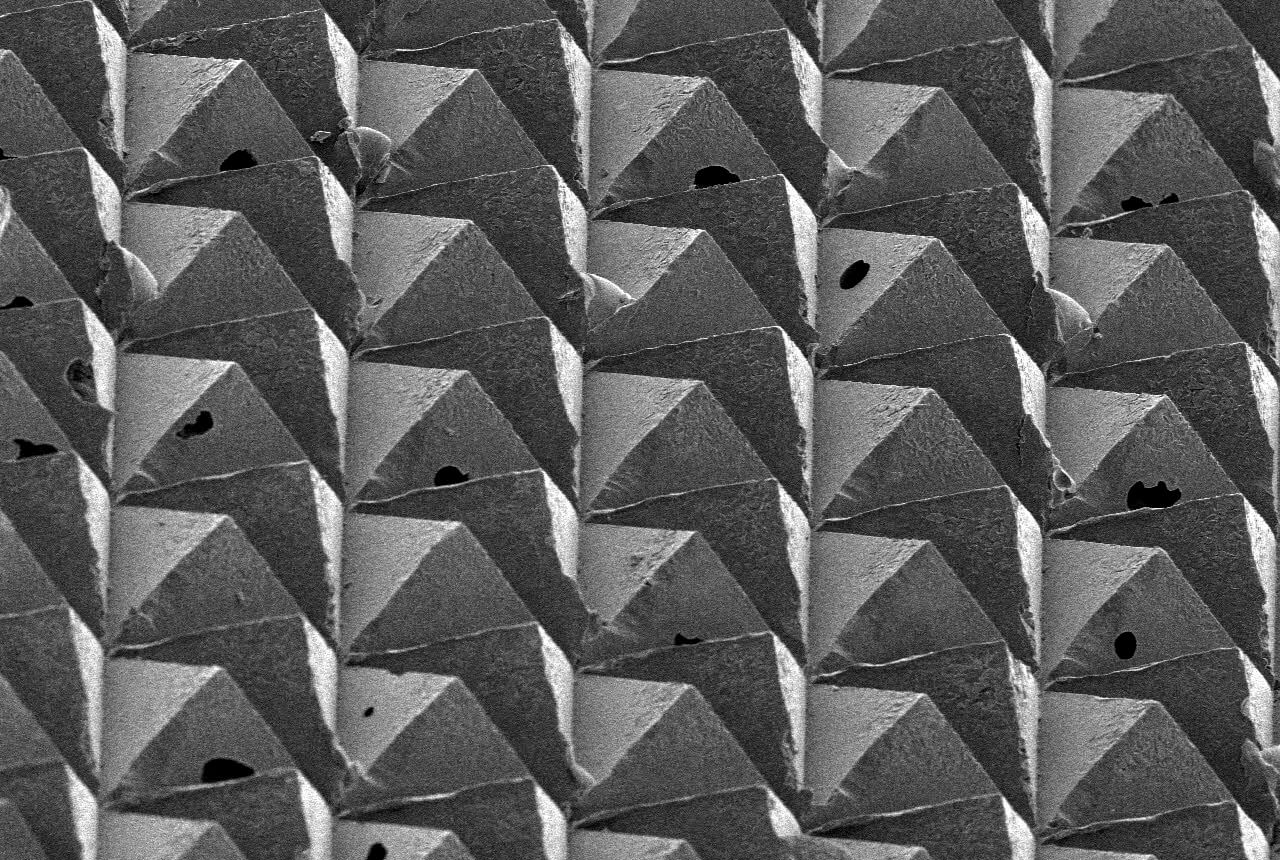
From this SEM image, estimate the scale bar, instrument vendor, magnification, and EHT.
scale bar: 100000 nm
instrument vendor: JEOL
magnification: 0.2 K X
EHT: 5 kV